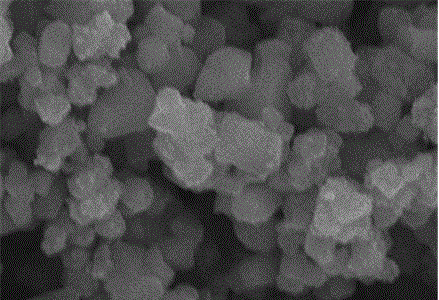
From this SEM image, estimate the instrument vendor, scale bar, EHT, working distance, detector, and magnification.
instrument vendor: Hitachi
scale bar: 2000 nm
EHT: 15 kV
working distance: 5.9 mm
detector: SE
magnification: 20 K X